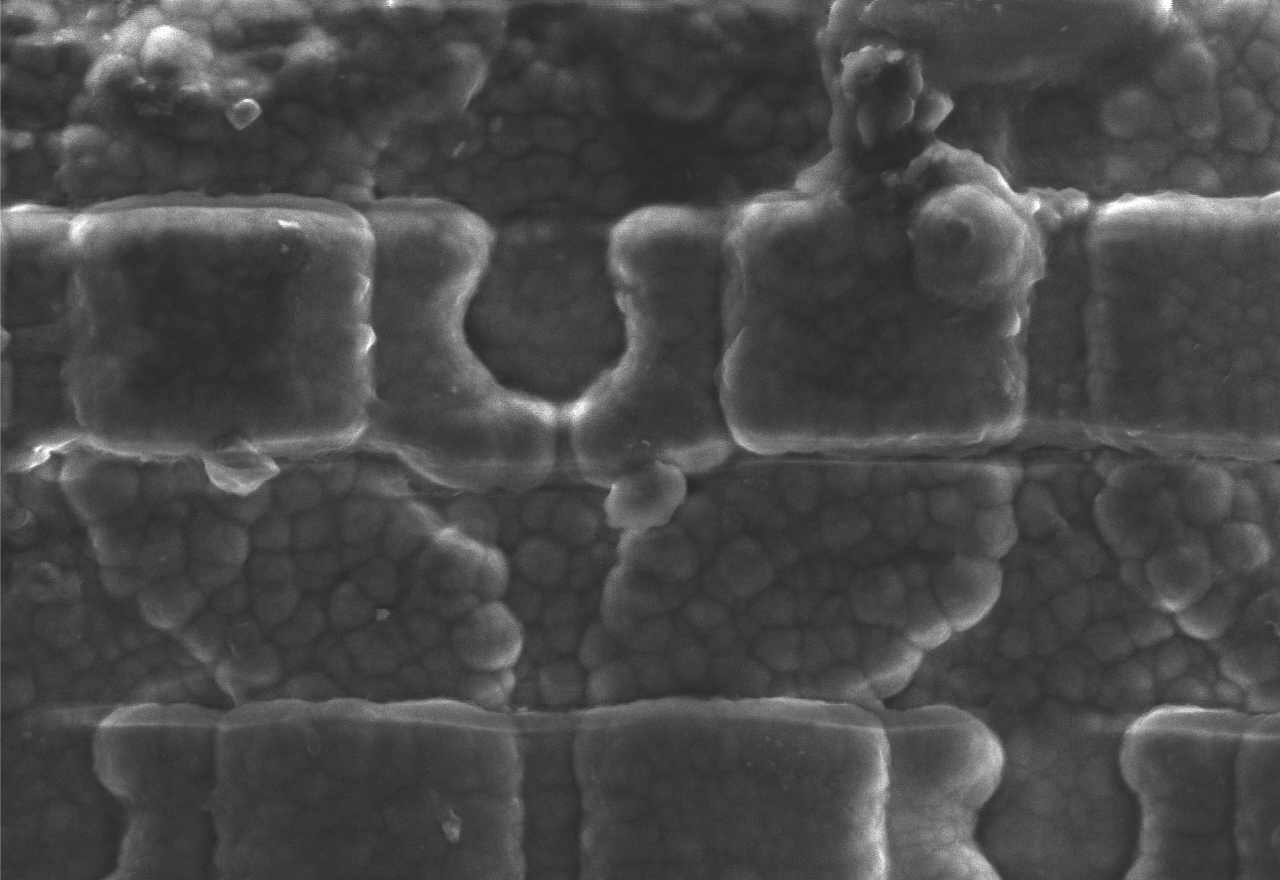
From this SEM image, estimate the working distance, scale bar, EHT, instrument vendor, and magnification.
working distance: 8.5 mm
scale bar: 2850 nm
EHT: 8 kV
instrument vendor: other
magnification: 3.5 K X